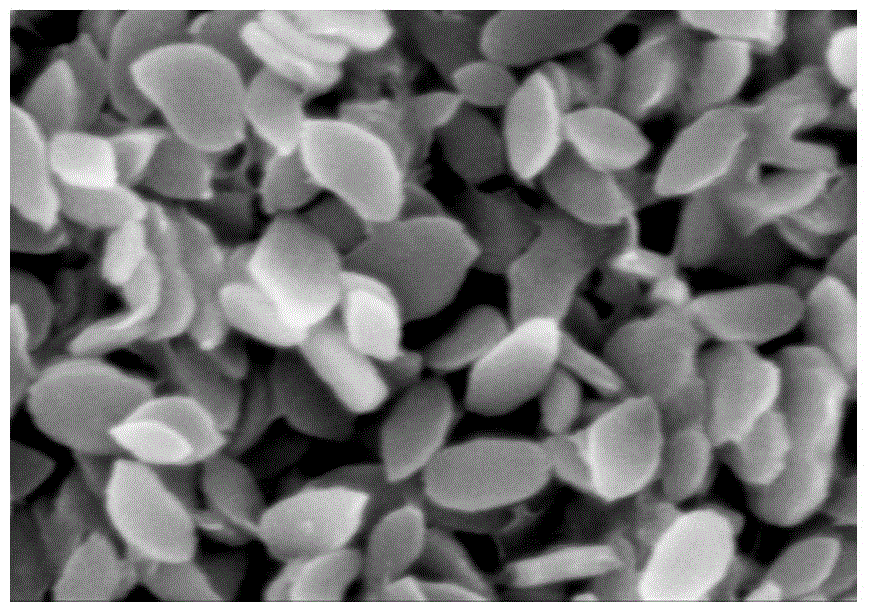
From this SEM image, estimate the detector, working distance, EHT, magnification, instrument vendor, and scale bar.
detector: SE(M)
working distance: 7.9 mm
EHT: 5 kV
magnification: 100 K X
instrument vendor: Hitachi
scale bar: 500 nm